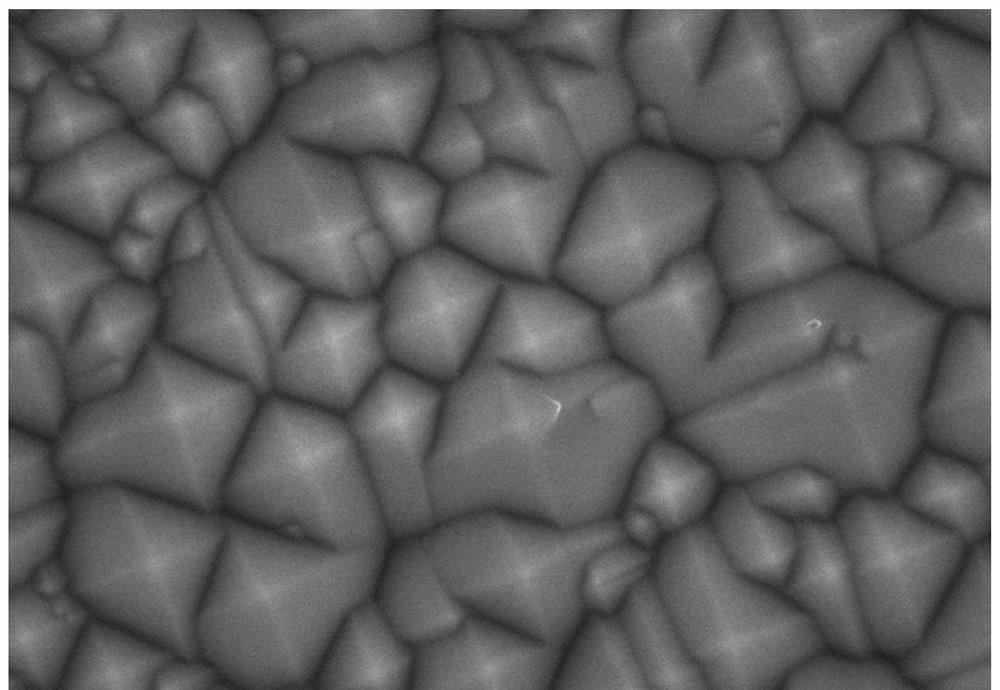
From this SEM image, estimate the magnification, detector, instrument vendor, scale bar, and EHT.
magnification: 10 K X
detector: SE(U)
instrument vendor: Hitachi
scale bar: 5000 nm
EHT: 10 kV